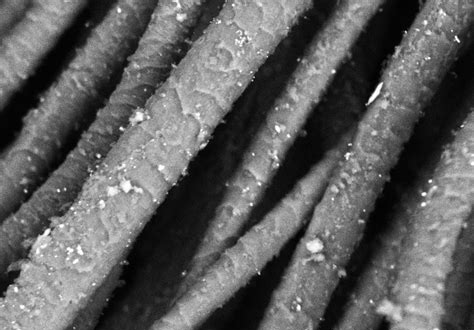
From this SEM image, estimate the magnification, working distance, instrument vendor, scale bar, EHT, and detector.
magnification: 0.5 K X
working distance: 10 mm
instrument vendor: Hitachi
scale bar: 100000 nm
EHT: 20 kV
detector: BSECOMP